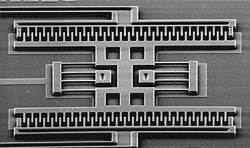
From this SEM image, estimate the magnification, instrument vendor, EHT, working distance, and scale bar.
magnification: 0.6 K X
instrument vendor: JEOL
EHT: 10 kV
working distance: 48 mm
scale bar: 10000 nm